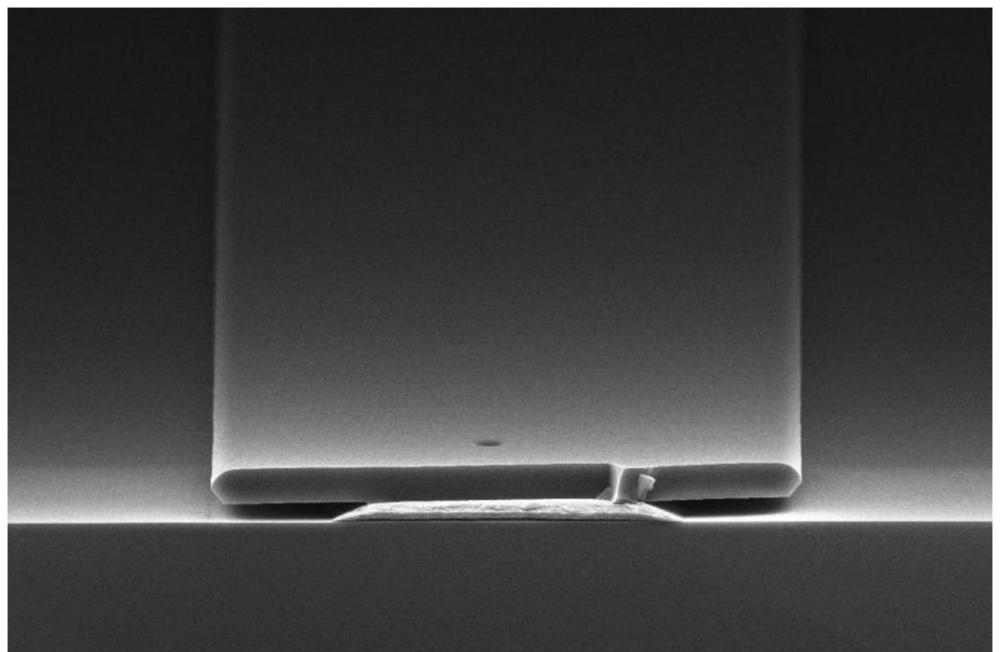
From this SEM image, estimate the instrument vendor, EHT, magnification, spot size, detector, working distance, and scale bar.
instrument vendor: Thermo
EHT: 15 kV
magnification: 2.5 K X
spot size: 7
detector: T2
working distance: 8.9073 mm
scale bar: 30000 nm